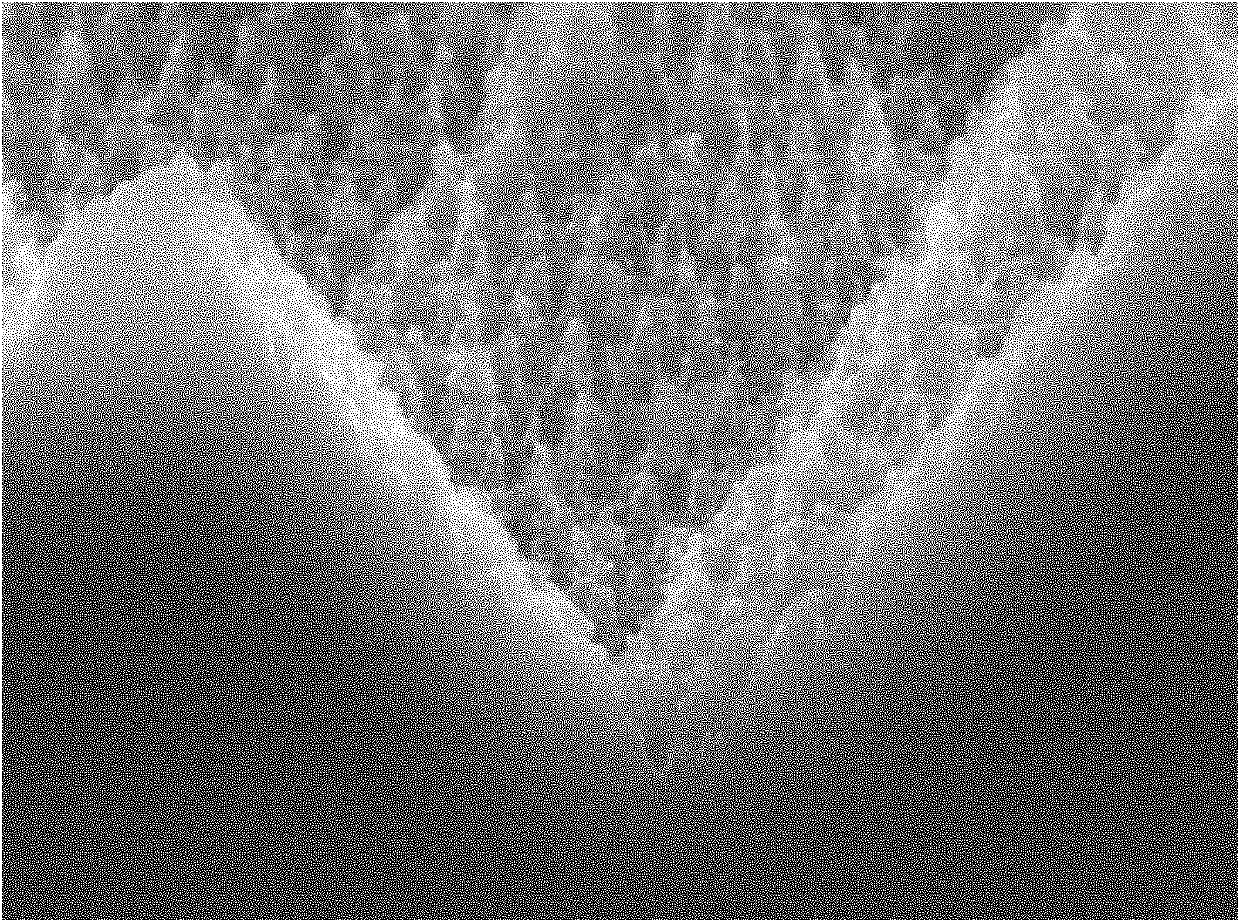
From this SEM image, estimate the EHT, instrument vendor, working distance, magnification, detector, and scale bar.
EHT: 10 kV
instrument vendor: JEOL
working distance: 6 mm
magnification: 20 K X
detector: SEI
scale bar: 1000 nm